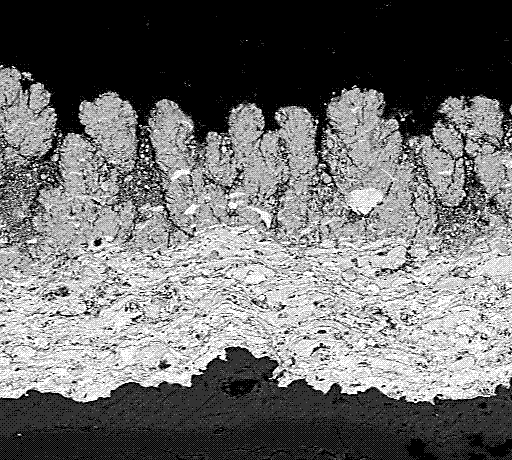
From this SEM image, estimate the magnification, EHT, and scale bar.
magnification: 0.35 K X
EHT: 25 kV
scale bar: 50000 nm